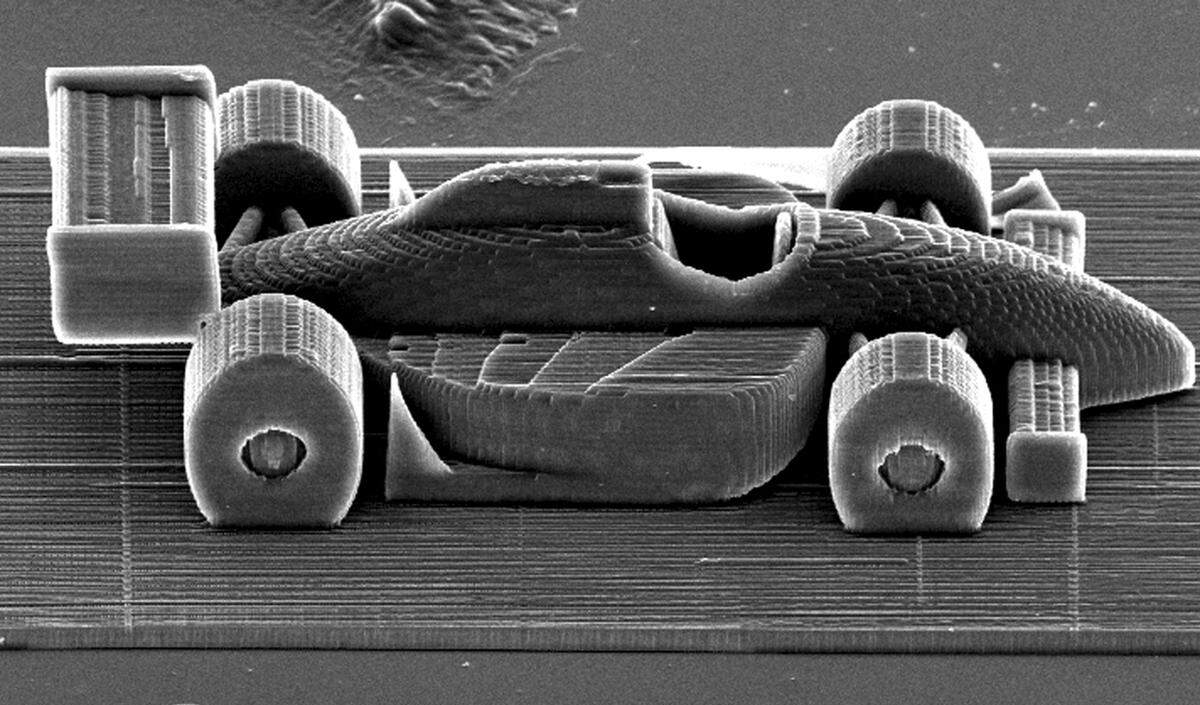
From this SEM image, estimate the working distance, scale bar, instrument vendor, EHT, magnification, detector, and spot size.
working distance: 27.2 mm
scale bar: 100000 nm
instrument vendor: FEI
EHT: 20 kV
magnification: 0.363 K X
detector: SE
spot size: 4